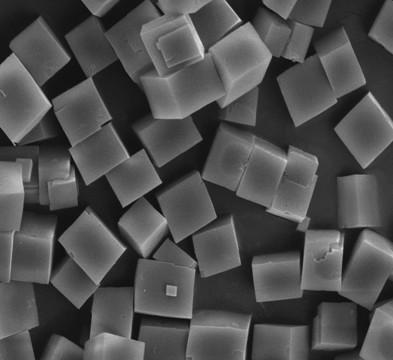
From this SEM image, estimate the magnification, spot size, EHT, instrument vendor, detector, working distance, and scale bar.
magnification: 10 K X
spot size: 3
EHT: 10 kV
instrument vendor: FEI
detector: ETD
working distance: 4.5 mm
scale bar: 5000 nm